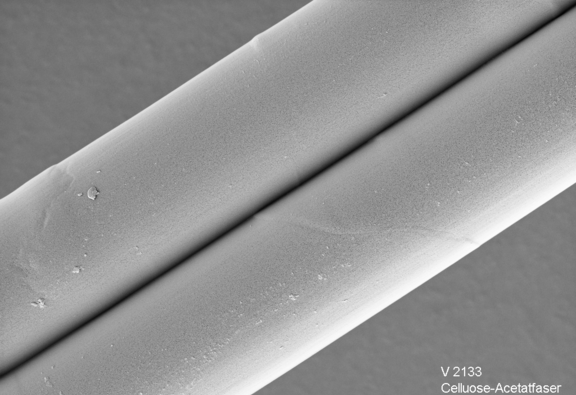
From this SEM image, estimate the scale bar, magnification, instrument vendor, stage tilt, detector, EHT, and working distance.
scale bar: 2000 nm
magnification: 5.54 K X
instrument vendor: Zeiss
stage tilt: -0°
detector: SE2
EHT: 3 kV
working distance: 8.3 mm